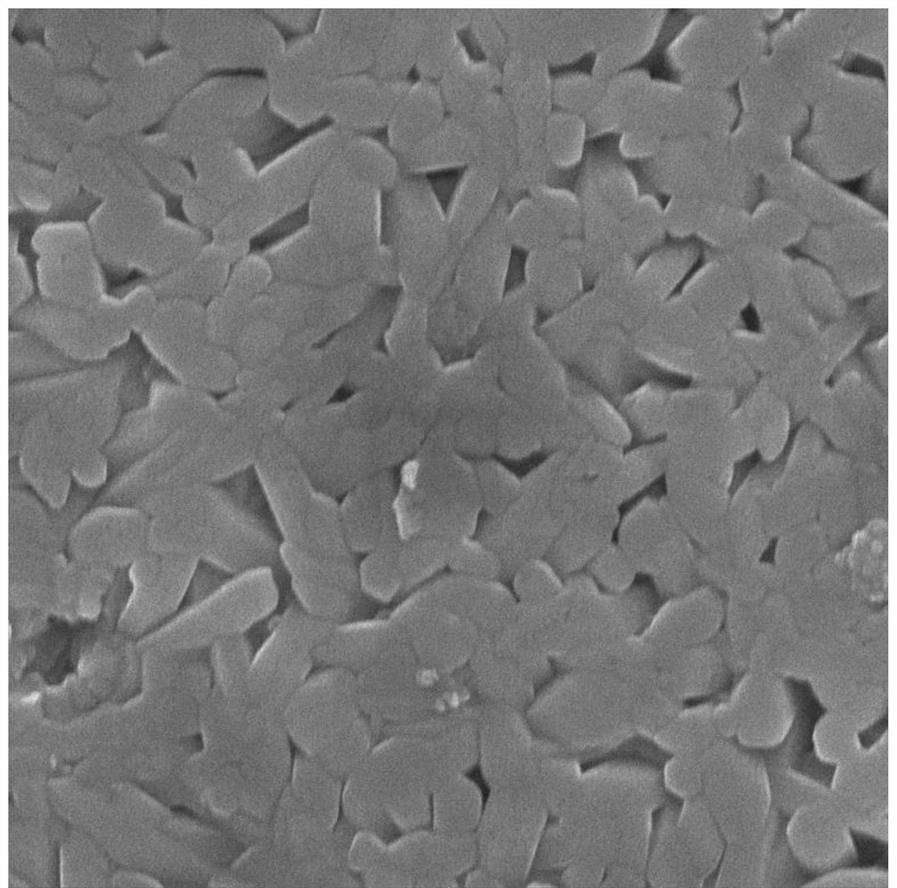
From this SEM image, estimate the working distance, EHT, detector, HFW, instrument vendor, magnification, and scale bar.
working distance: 7.08 mm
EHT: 15 kV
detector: In-Beam SE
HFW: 4.15 µm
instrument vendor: Tescan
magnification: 50 K X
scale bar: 1000 nm